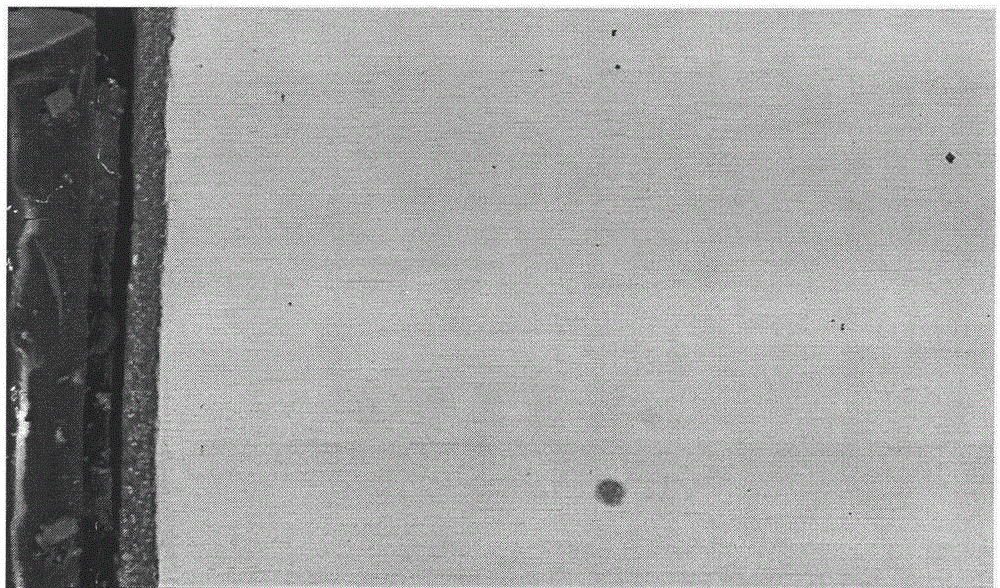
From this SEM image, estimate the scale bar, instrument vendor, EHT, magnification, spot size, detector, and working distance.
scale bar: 5000 nm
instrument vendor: FEI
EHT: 15 kV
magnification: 4 K X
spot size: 5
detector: BSE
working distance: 7.4 mm